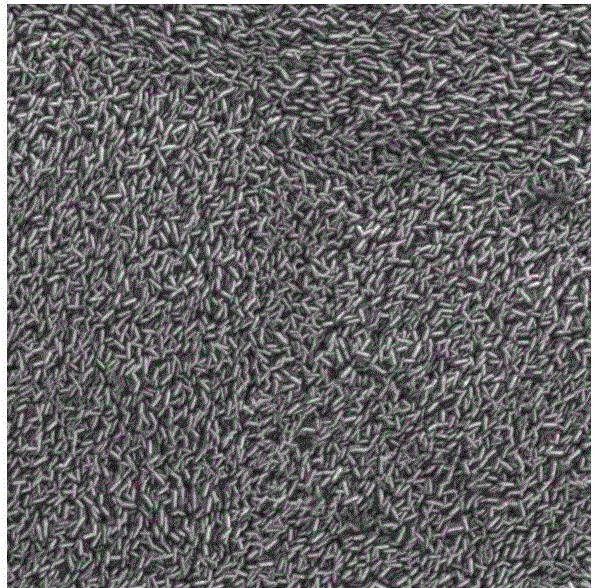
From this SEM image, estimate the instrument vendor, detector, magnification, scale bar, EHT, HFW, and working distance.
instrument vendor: Tescan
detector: SE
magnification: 10 K X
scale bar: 5000 nm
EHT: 20 kV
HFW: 20.8 µm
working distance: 14.54 mm